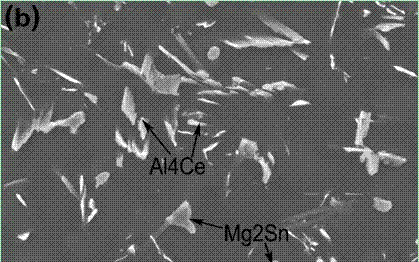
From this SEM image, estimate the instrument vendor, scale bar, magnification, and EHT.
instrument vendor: JEOL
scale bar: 10000 nm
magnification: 1 K X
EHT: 20 kV